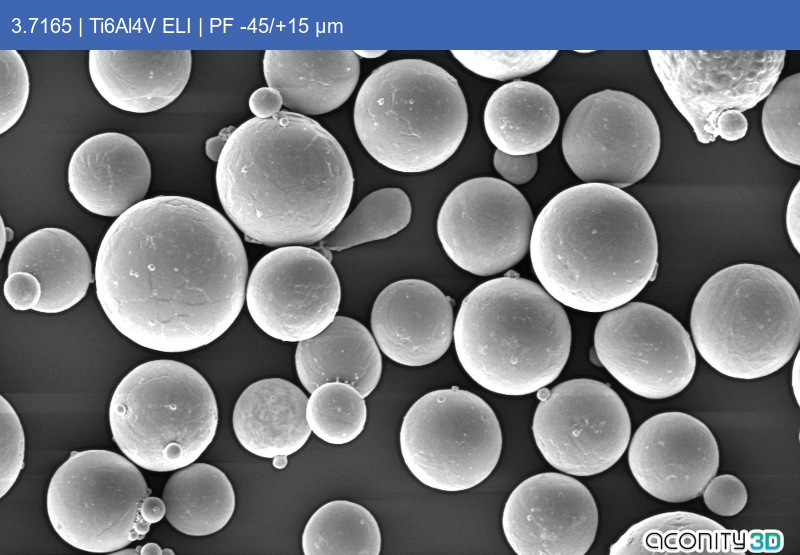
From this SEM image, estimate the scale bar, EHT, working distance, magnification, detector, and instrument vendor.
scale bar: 20000 nm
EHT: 10 kV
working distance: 10.97 mm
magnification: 0.5 K X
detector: SE1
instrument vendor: Zeiss